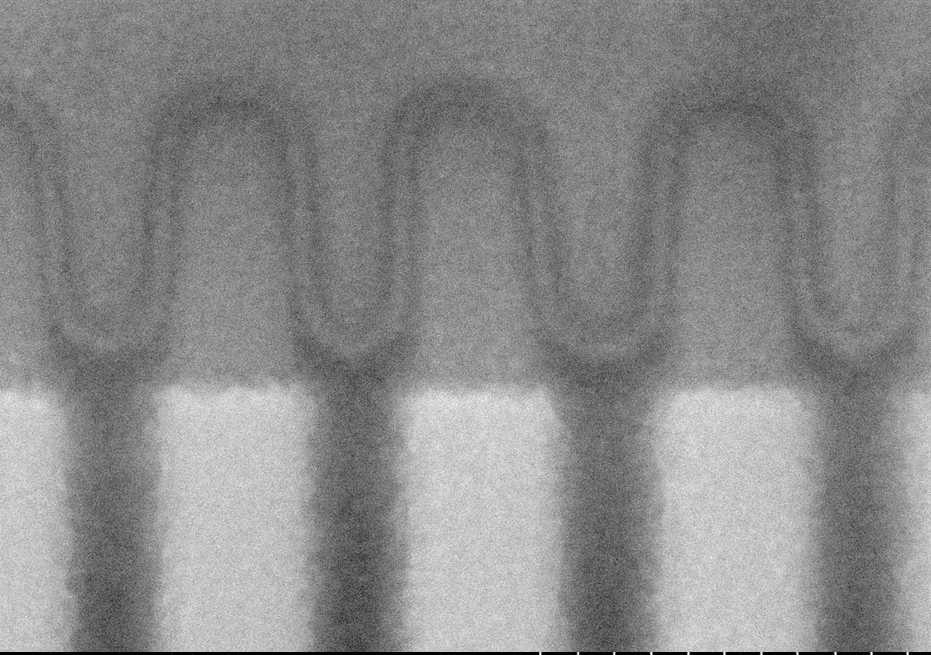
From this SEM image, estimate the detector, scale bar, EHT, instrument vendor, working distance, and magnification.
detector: LA-BSE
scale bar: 100 nm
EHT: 3 kV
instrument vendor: Hitachi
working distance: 2 mm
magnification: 500 K X